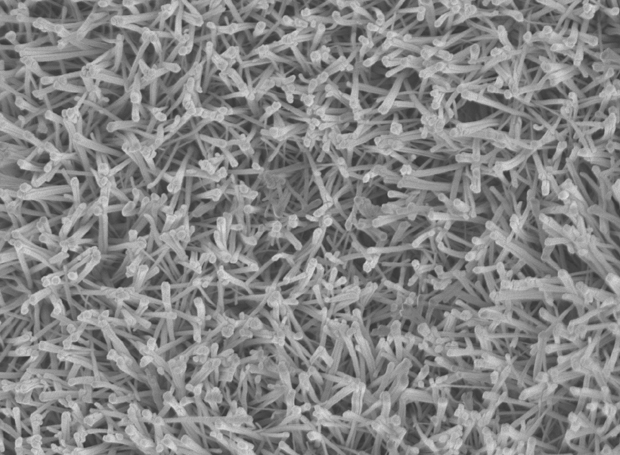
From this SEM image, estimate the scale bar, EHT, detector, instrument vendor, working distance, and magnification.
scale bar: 1000 nm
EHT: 5 kV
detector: SEI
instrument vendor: JEOL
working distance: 6 mm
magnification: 10 K X